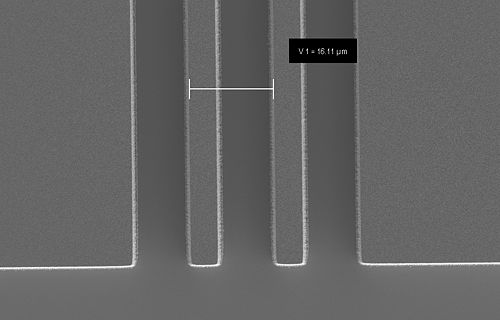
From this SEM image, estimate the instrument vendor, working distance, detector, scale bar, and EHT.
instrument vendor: Zeiss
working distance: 10.7 mm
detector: SE2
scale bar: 10000 nm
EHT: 2.5 kV